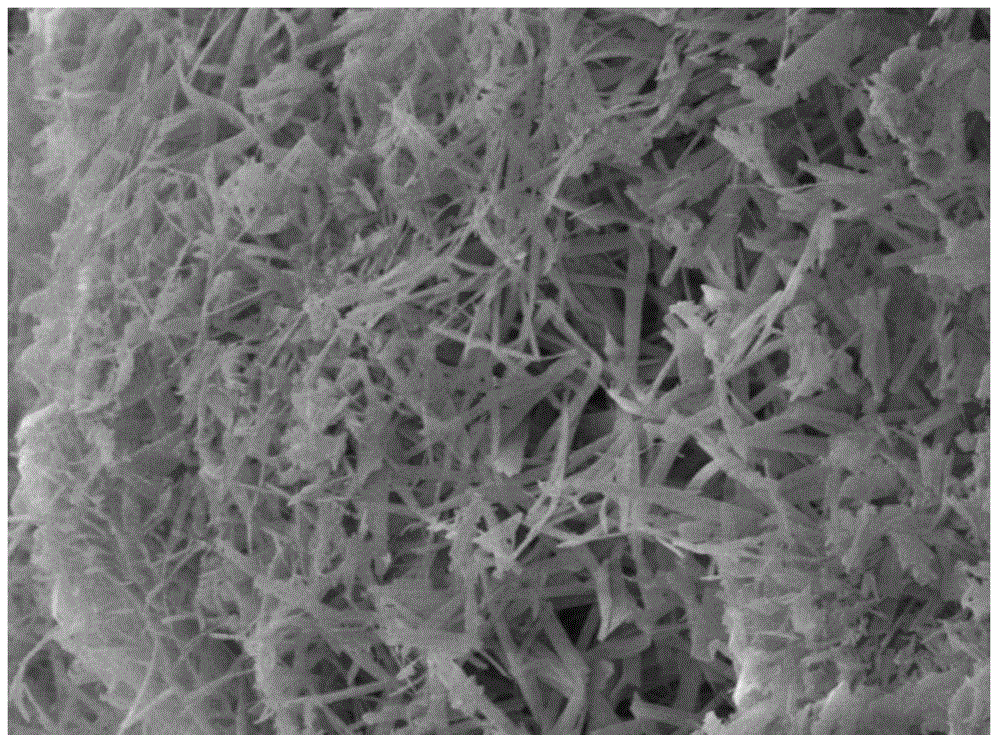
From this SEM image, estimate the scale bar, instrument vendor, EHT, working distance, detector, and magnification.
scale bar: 1000 nm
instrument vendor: JEOL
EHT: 5 kV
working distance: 9.9 mm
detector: SEI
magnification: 10 K X